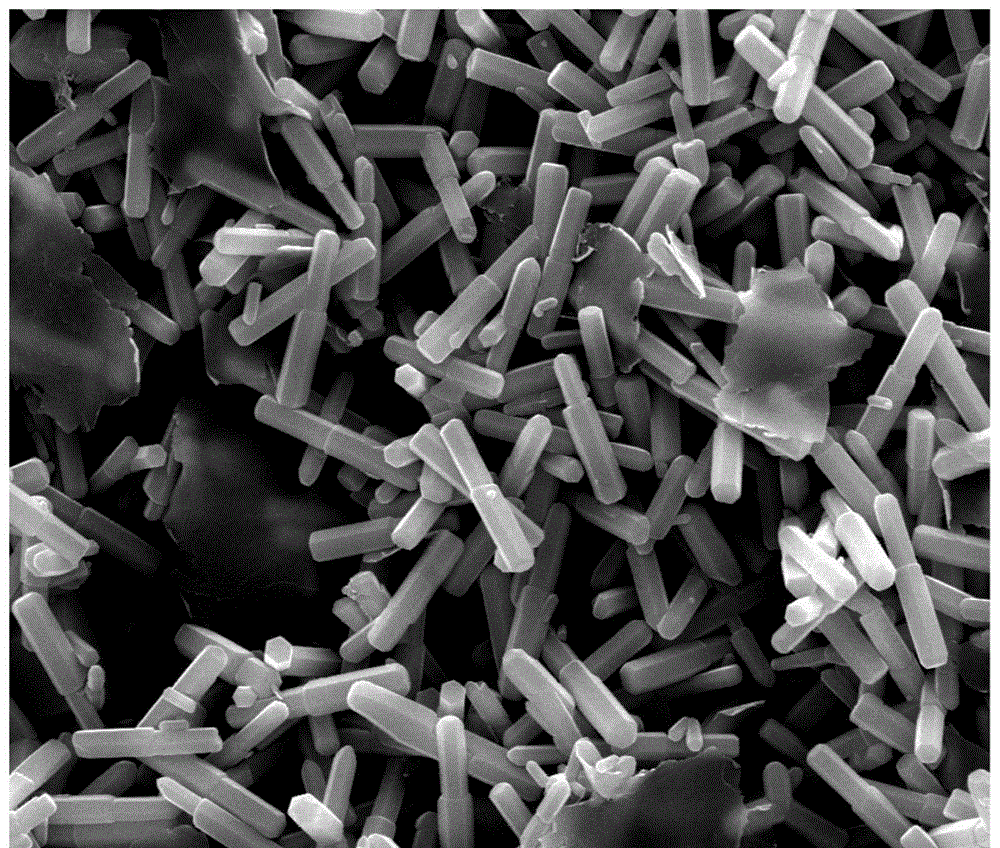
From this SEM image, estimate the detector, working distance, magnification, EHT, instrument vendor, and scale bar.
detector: SE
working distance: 10.1 mm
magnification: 20 K X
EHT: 20 kV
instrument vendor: FEI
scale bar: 5000 nm